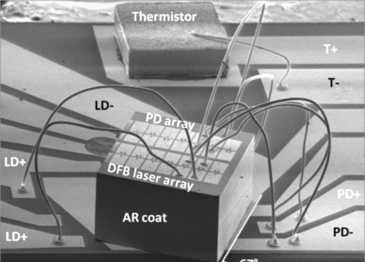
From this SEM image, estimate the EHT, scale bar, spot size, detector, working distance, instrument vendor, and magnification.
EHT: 5 kV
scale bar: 1000000 nm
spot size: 3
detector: SE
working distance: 3.9 mm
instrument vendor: FEI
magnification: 0.037 K X